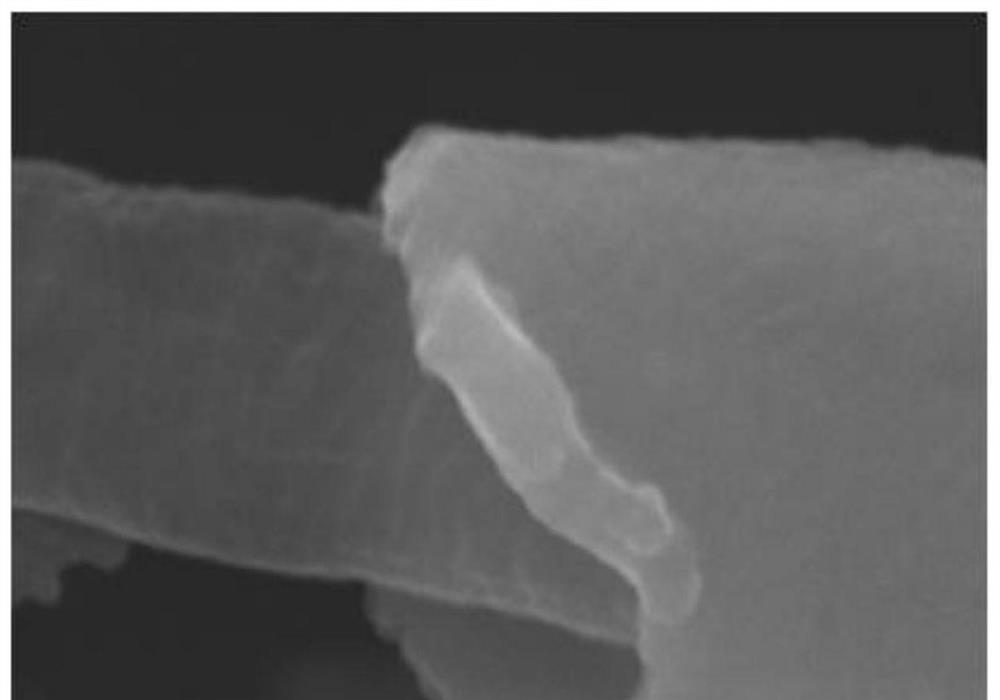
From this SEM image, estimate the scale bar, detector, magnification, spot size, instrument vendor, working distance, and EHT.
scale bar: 500 nm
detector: SEI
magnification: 50 K X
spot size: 30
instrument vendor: JEOL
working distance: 12 mm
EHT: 30 kV